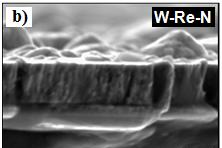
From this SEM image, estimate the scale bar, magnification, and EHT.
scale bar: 2000 nm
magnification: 7.5 K X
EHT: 20 kV